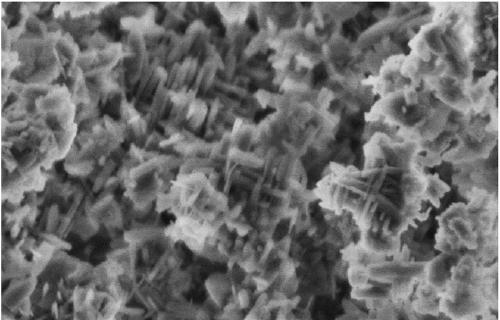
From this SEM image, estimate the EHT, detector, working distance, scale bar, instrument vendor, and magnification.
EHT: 2 kV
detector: SE(M)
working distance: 8.5 mm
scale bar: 1000 nm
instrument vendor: Hitachi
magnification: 40 K X